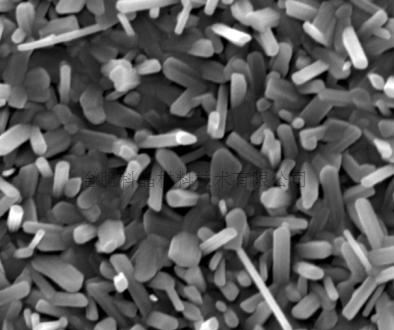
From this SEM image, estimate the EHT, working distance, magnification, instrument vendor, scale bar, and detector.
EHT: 20 kV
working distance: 6 mm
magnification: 40 K X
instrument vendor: FEI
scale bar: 1000 nm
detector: TLD